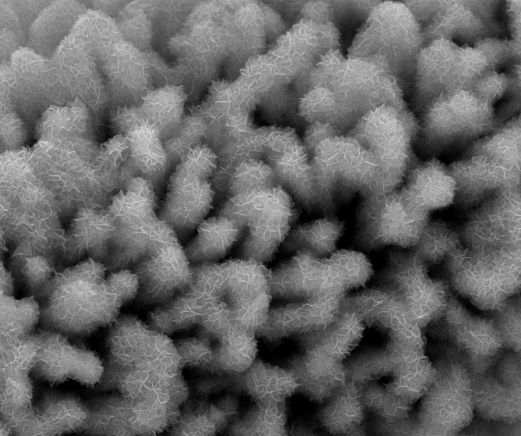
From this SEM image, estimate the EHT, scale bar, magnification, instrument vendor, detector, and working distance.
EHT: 20 kV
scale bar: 5000 nm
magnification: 10 K X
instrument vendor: FEI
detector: ETD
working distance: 10 mm